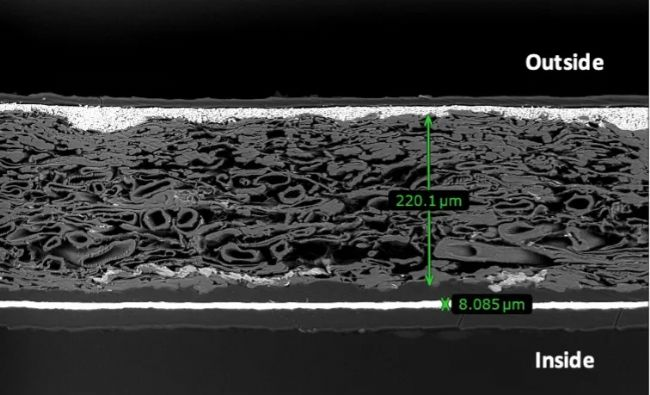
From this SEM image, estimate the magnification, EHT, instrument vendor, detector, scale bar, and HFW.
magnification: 0.15 K X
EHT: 15 kV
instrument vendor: Thermo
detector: BSD Full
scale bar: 200000 nm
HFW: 847 µm